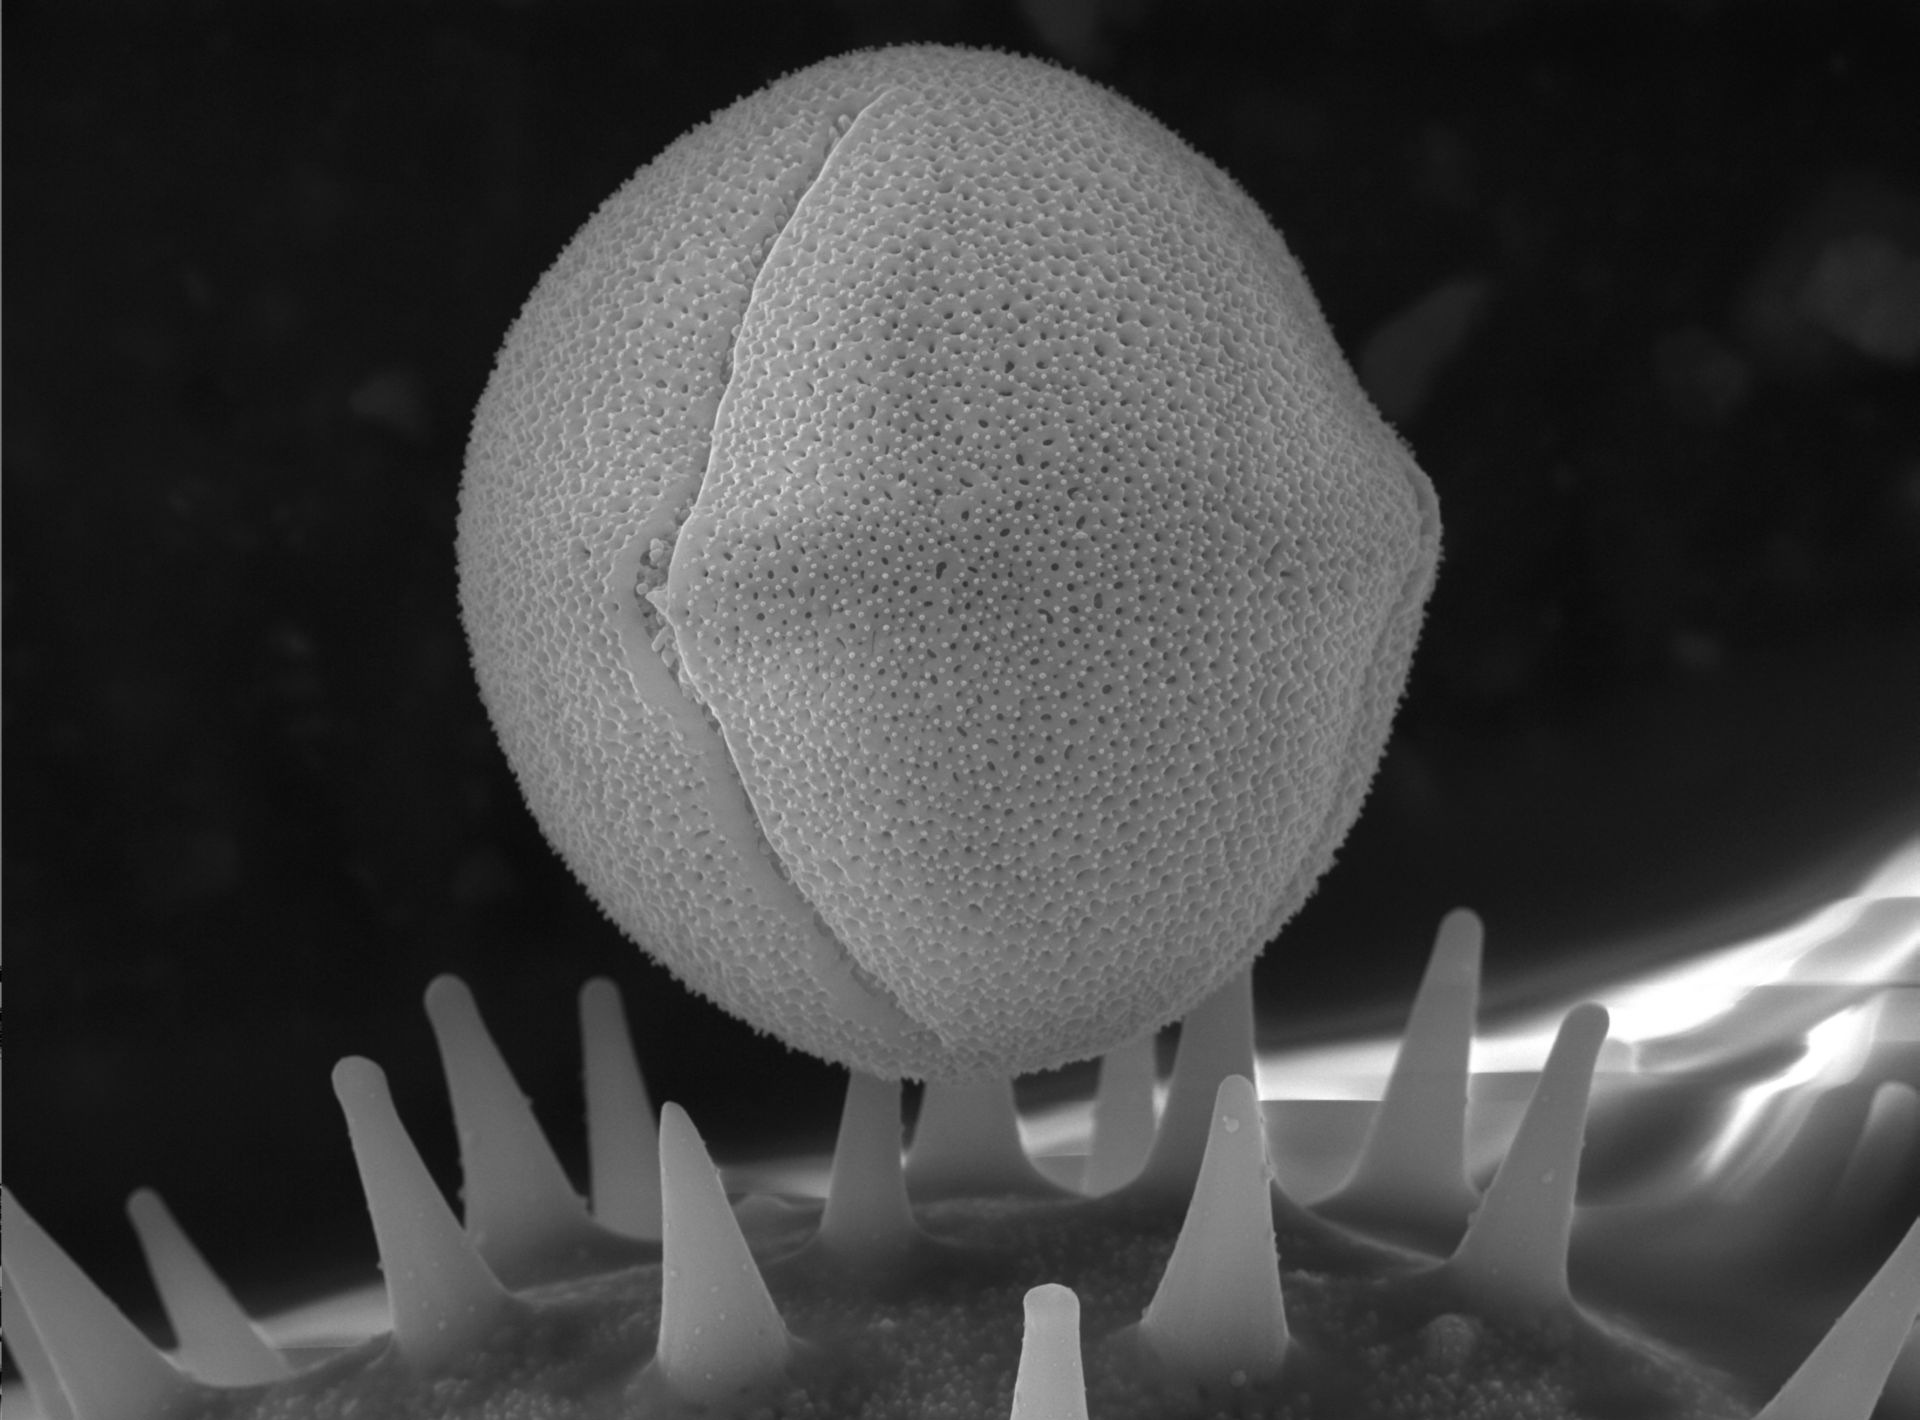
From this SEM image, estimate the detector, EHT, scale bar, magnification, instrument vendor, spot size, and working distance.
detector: NONE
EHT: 15 kV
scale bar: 10000 nm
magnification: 3 K X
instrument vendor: FEI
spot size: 3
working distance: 9.9 mm